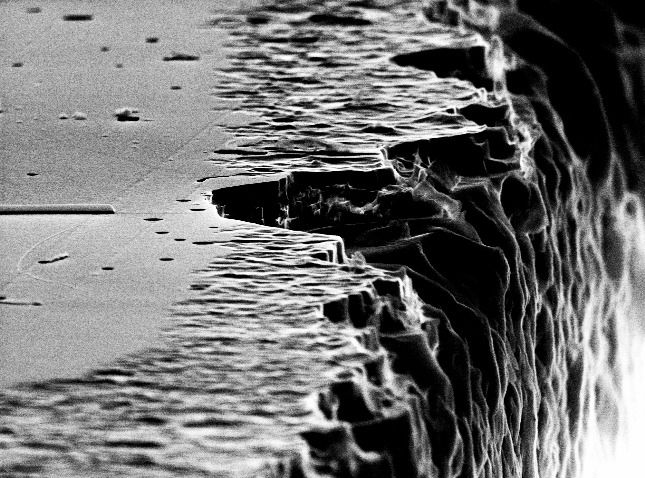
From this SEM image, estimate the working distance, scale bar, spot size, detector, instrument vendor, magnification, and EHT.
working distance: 18.6 mm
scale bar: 2000 nm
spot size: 3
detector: SE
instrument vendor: FEI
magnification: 14.62 K X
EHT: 30 kV